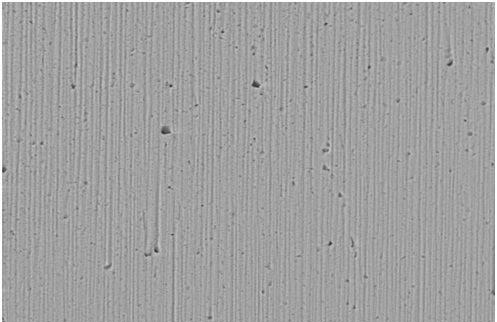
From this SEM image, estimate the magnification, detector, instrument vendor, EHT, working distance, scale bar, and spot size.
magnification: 1 K X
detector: BSE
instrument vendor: FEI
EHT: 20 kV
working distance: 10 mm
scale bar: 20000 nm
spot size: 5